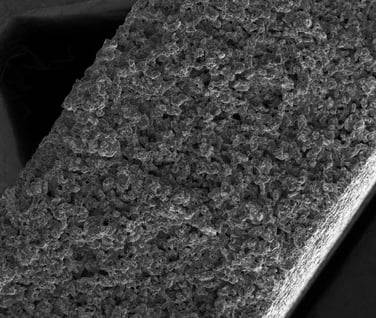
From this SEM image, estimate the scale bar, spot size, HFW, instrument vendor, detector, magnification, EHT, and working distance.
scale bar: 500000 nm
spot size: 2.5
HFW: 2230 µm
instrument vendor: FEI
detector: ETD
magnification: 0.067 K X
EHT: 7 kV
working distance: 6.1 mm